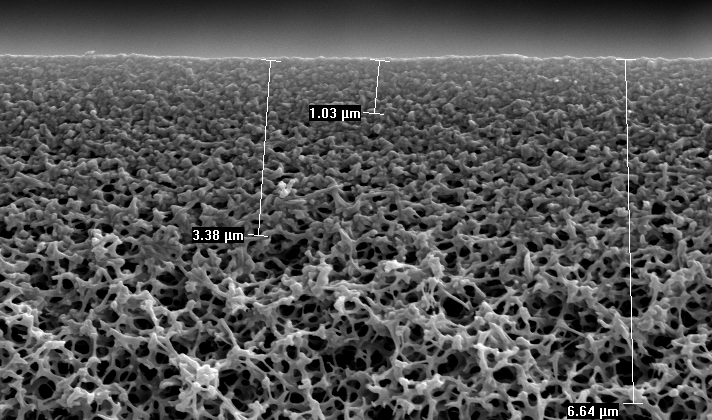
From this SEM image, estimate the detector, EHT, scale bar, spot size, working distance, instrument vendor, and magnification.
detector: SE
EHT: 10 kV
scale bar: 2000 nm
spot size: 1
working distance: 6.6 mm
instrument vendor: FEI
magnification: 10 K X